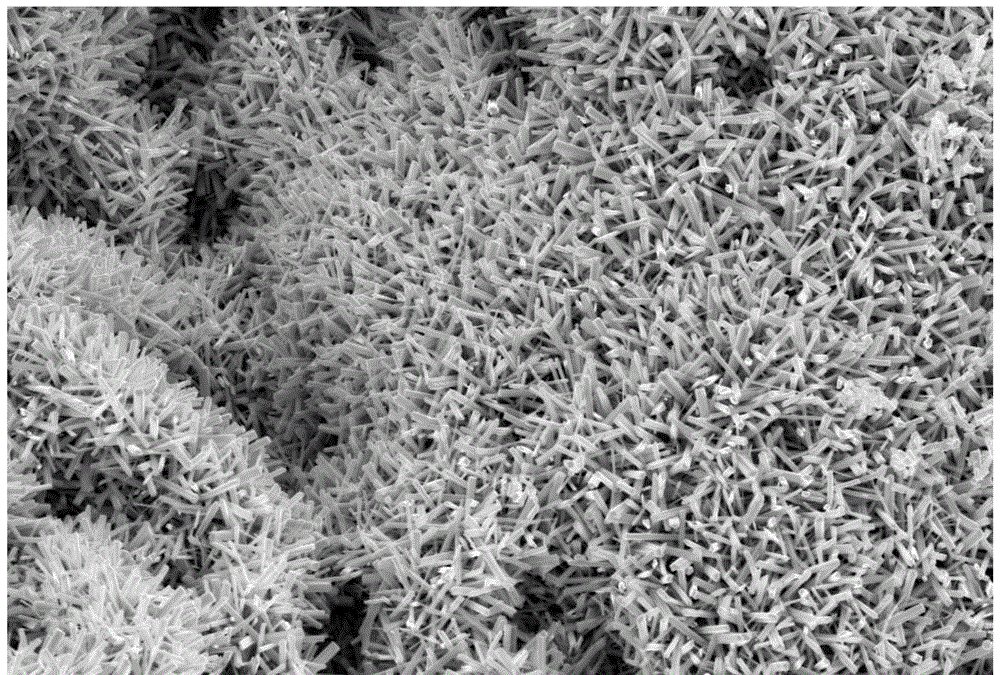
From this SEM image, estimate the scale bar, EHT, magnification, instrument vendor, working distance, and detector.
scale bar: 2000 nm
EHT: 10 kV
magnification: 3 K X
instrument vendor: Zeiss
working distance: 7.3 mm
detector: InLens